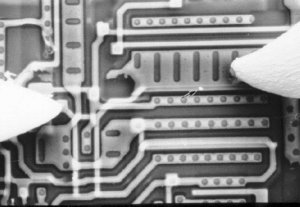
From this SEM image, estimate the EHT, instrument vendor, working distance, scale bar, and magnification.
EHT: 20 kV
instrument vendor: JEOL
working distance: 45 mm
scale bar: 10000 nm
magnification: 1.7 K X